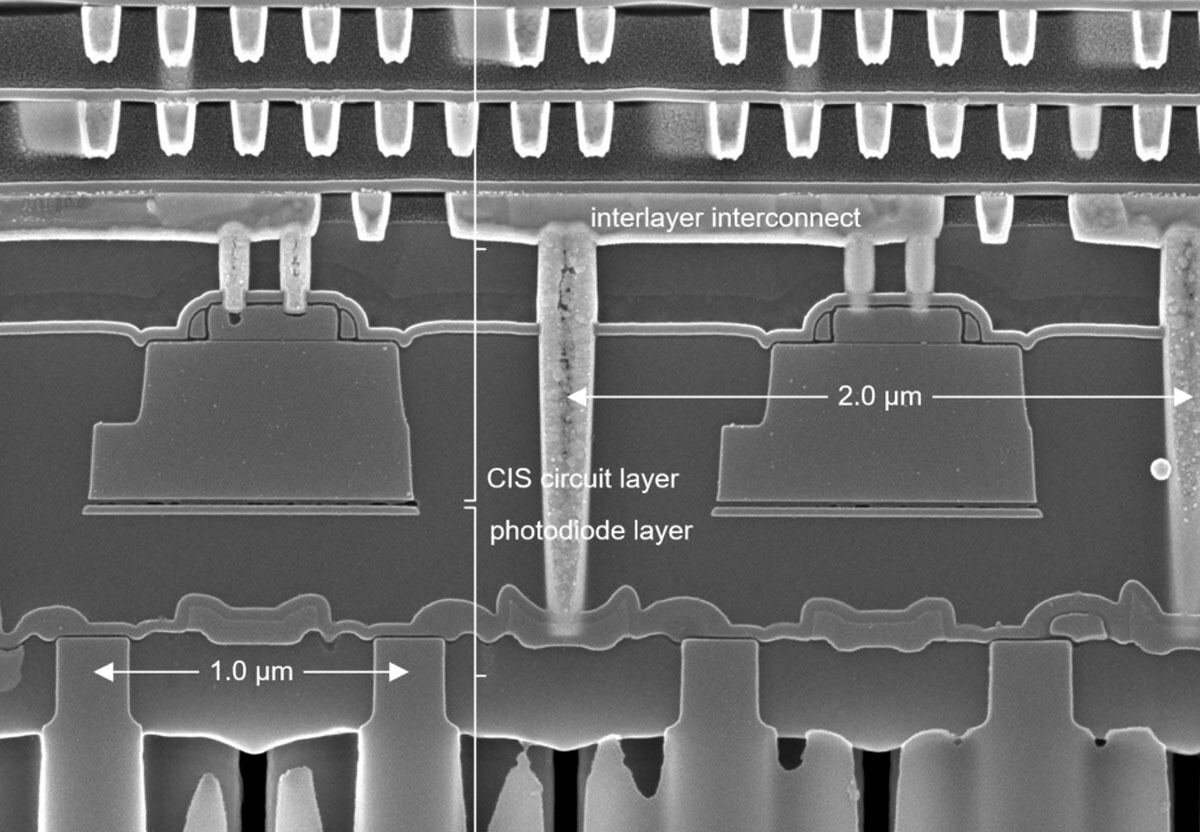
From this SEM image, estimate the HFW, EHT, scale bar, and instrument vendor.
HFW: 3.826 µm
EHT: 5 kV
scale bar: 200 nm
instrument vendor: Zeiss